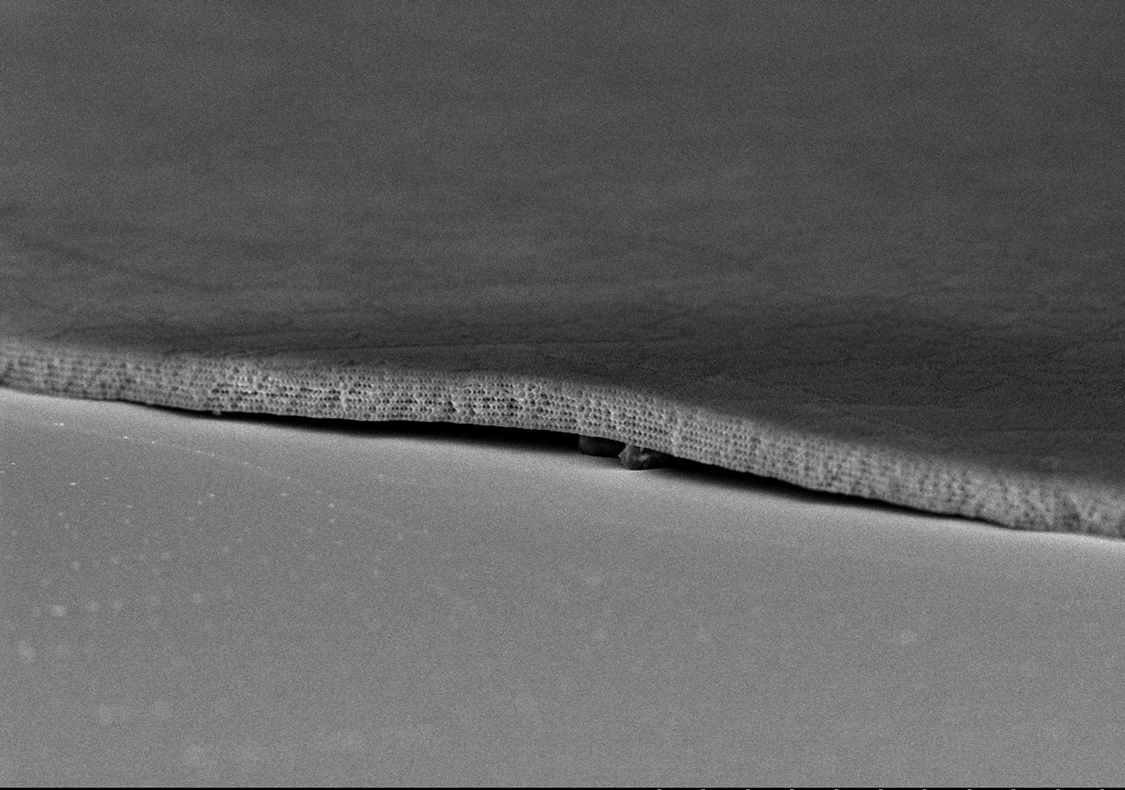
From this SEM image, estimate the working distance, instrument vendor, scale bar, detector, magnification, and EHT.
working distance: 8.7 mm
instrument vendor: Hitachi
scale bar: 20000 nm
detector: SE(U)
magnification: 2.5 K X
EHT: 5 kV